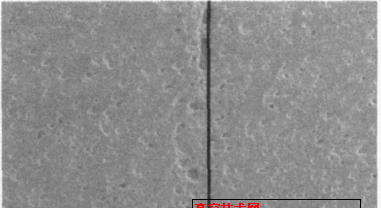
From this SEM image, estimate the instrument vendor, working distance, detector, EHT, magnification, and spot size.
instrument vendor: FEI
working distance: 12.8 mm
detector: SE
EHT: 20 kV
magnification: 0.1 K X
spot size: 4.2